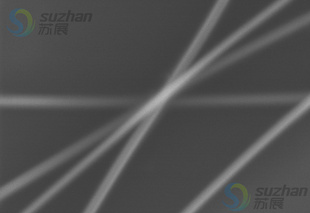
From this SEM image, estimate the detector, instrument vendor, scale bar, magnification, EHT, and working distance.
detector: SE(M)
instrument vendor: Hitachi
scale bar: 500 nm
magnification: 60 K X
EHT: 5 kV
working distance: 8 mm